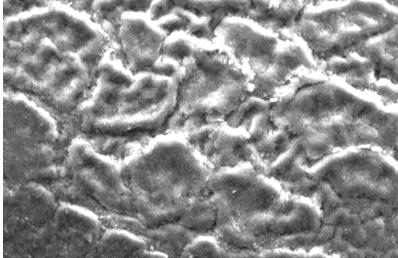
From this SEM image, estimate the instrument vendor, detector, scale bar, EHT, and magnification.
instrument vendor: JEOL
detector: SEI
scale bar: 10000 nm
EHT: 20 kV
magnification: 1 K X